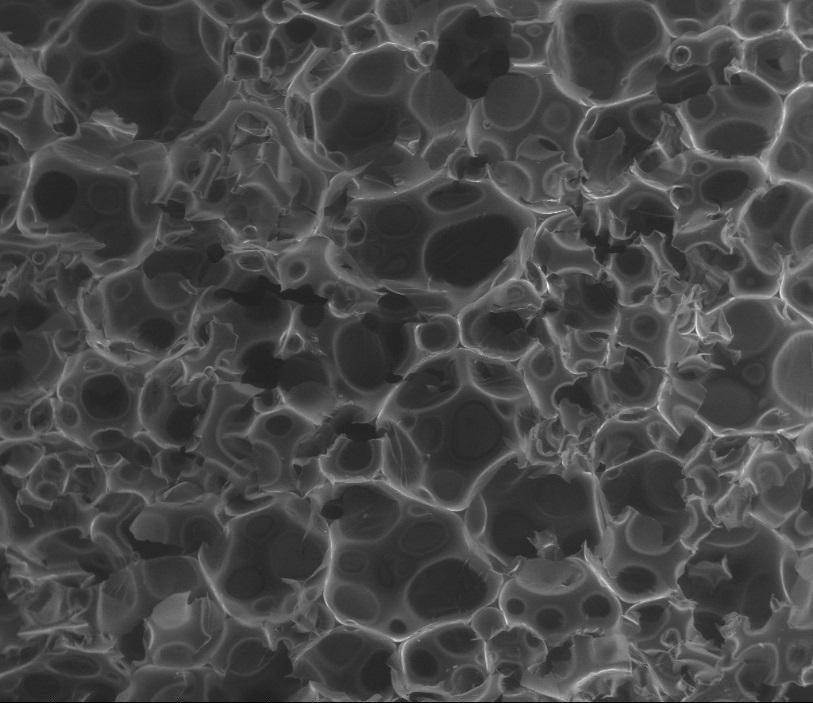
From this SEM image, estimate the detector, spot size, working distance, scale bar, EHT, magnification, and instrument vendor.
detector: LFD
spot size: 4.5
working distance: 13 mm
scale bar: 1000000 nm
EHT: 20 kV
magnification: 0.05 K X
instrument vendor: FEI